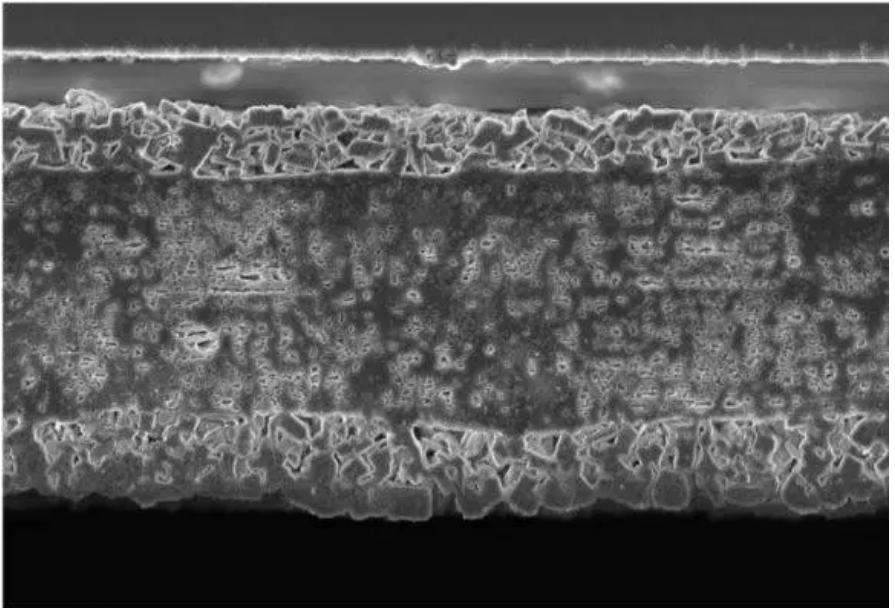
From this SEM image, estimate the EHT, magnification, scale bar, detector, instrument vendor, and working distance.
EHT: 3 kV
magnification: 5 K X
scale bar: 30000 nm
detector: InLens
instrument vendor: Zeiss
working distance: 2.3 mm